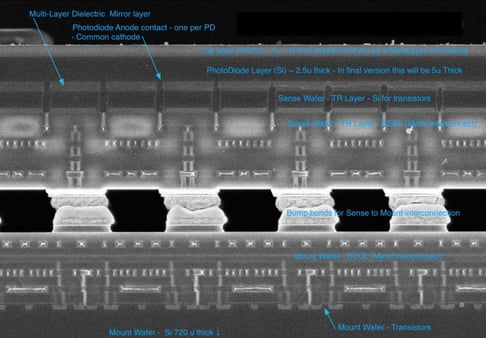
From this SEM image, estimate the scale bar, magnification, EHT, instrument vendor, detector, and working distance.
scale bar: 10000 nm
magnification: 3 K X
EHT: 10 kV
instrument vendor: Hitachi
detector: SE(U)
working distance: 7.7 mm